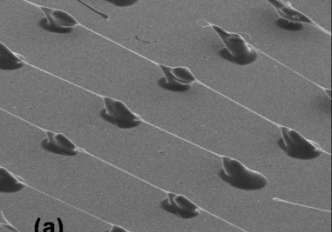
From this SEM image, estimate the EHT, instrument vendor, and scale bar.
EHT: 25 kV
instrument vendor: JEOL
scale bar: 500000 nm